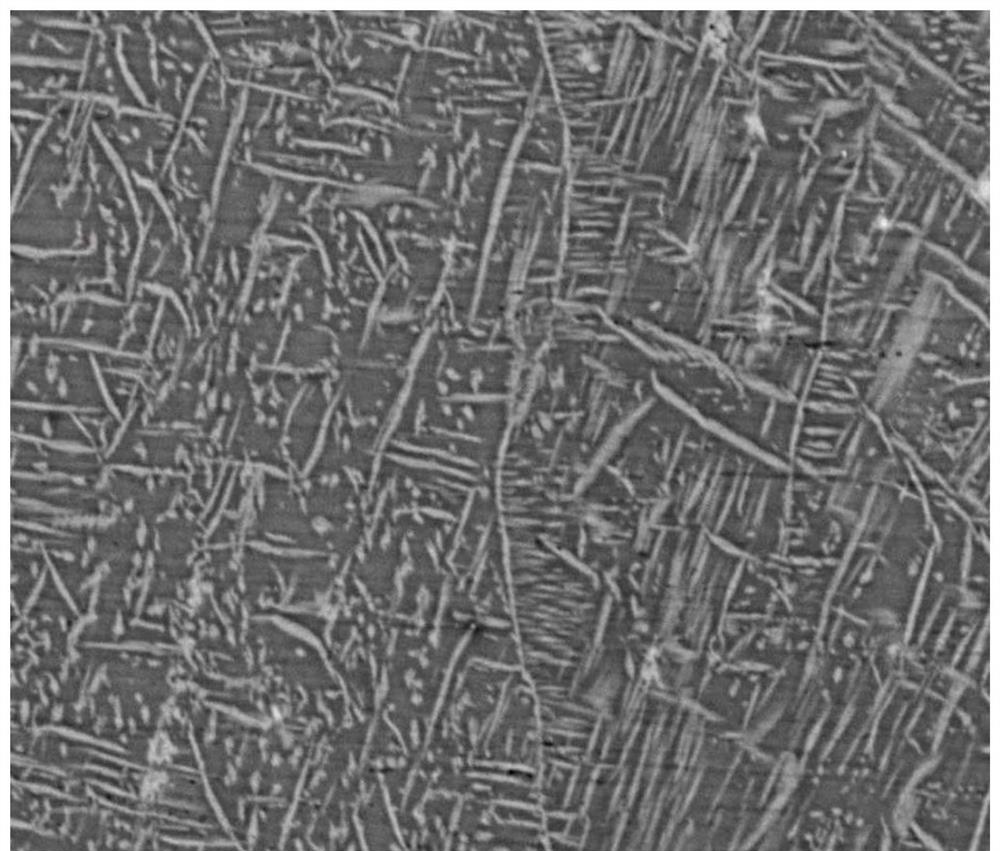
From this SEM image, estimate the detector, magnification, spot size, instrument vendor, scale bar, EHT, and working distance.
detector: DualBSD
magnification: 5 K X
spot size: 6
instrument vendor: FEI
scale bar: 20000 nm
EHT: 20 kV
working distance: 10 mm